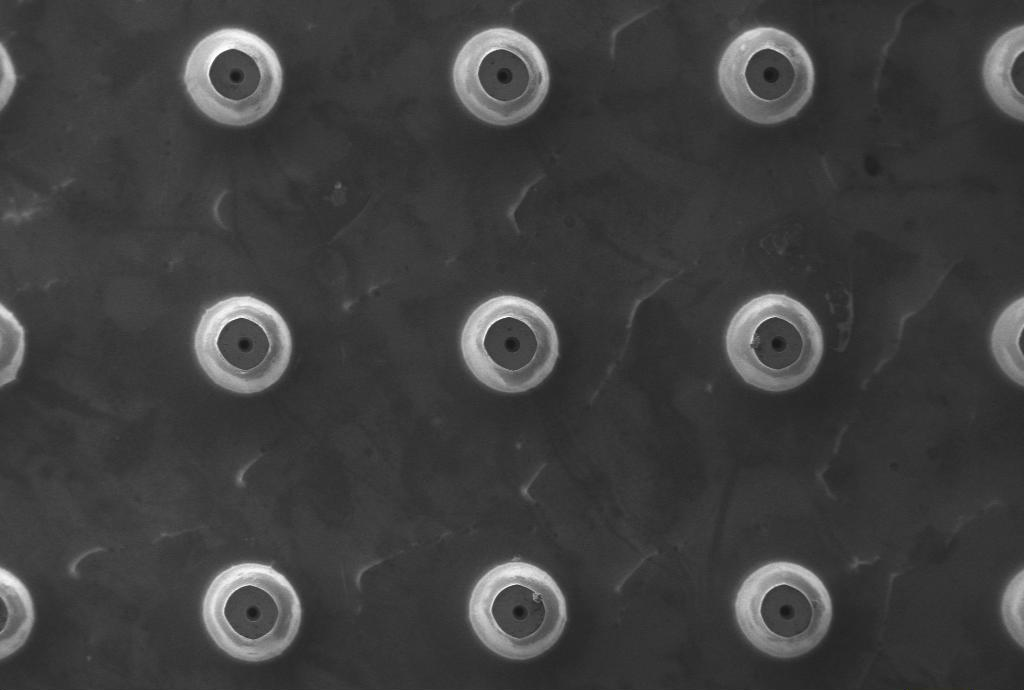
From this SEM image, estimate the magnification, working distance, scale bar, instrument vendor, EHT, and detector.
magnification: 0.06 K X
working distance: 6.5 mm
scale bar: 100000 nm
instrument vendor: Zeiss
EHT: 10 kV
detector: SE1